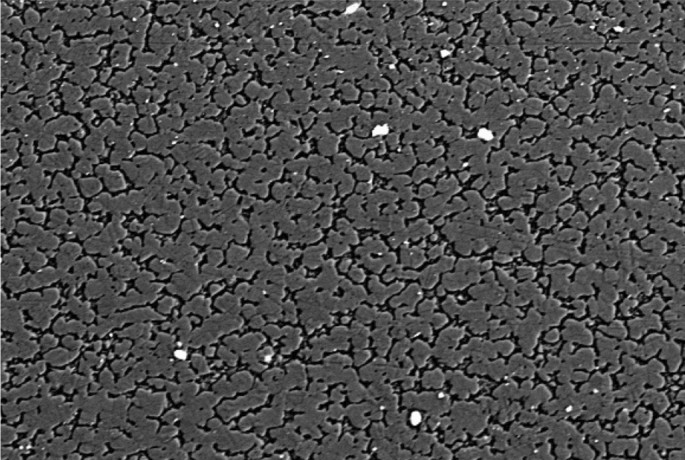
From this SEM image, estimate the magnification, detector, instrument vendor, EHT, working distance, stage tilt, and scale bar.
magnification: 2 K X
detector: NTS BSD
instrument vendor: Zeiss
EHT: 20 kV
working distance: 6 mm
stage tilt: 0°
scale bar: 10000 nm